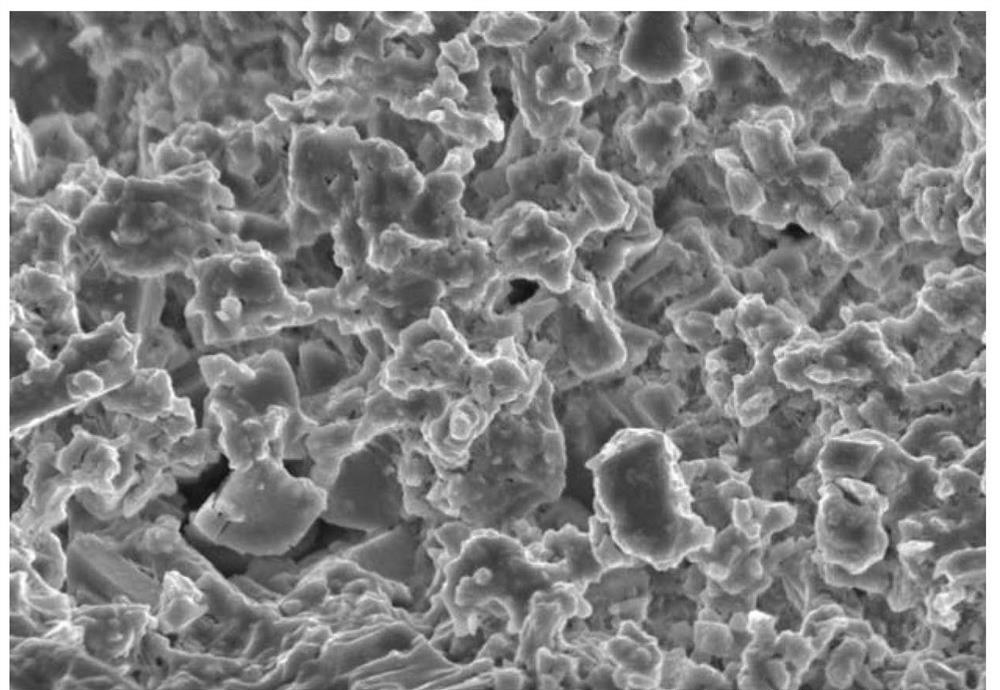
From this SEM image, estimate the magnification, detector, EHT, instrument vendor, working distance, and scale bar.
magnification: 2.1 K X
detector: SE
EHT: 15 kV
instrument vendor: Hitachi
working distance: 14.7 mm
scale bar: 20000 nm